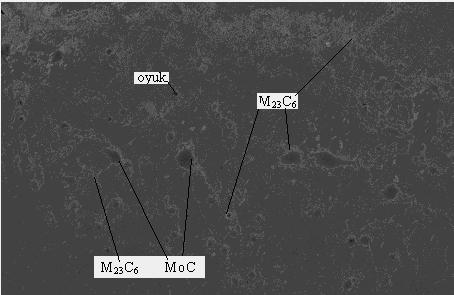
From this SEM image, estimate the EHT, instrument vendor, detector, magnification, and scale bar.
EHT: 20 kV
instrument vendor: Zeiss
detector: SE1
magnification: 0.3 K X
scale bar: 100000 nm